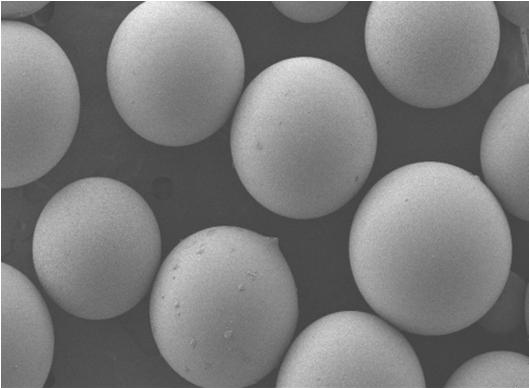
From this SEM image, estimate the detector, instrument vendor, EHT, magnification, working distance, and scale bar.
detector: LEI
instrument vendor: JEOL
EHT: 5 kV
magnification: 0.05 K X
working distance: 8.1 mm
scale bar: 100000 nm